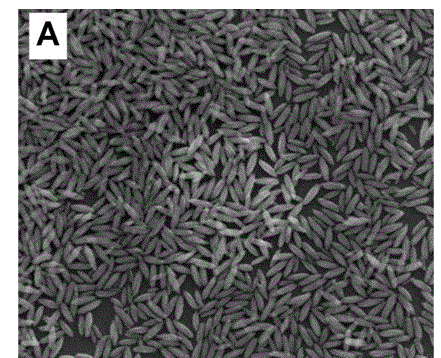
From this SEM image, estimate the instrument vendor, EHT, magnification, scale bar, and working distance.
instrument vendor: FEI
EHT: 10 kV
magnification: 80 K X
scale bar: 1000 nm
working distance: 15.3 mm